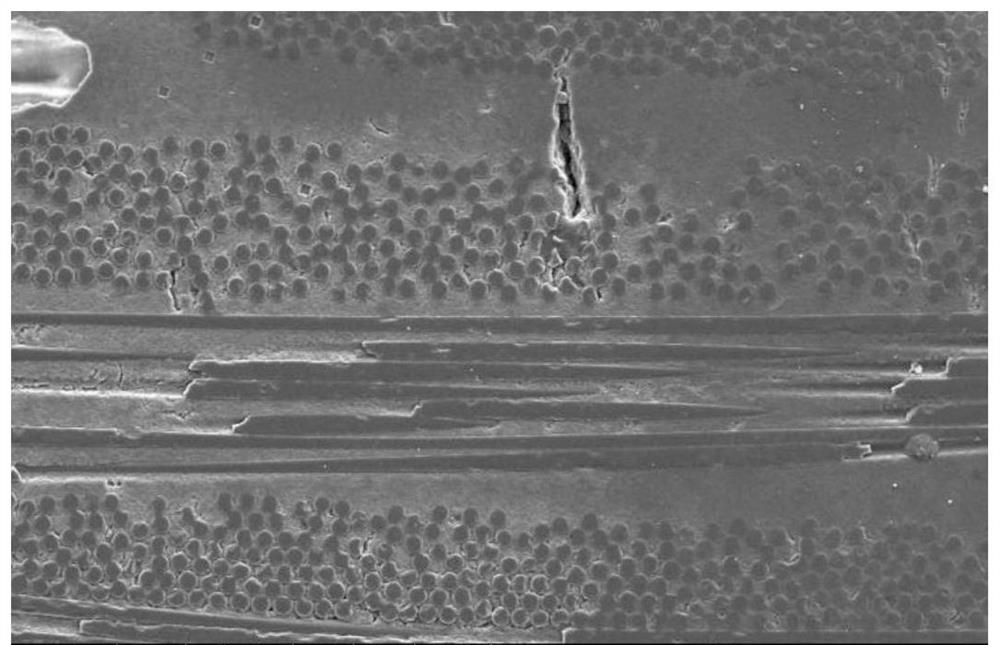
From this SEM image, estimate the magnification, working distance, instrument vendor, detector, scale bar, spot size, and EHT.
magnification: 0.5 K X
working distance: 11.3 mm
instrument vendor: FEI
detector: ETD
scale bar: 200000 nm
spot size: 5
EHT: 20 kV